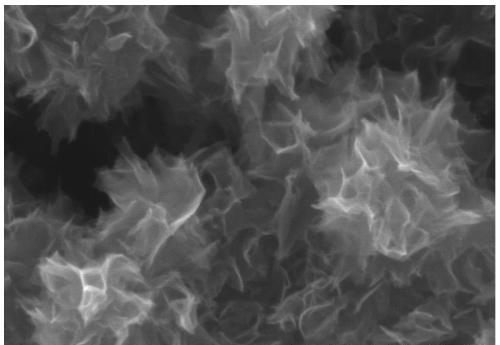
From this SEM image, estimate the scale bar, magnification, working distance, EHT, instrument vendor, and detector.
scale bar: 500 nm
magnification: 100 K X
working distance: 6.3 mm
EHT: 5 kV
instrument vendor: Hitachi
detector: SE(UL)